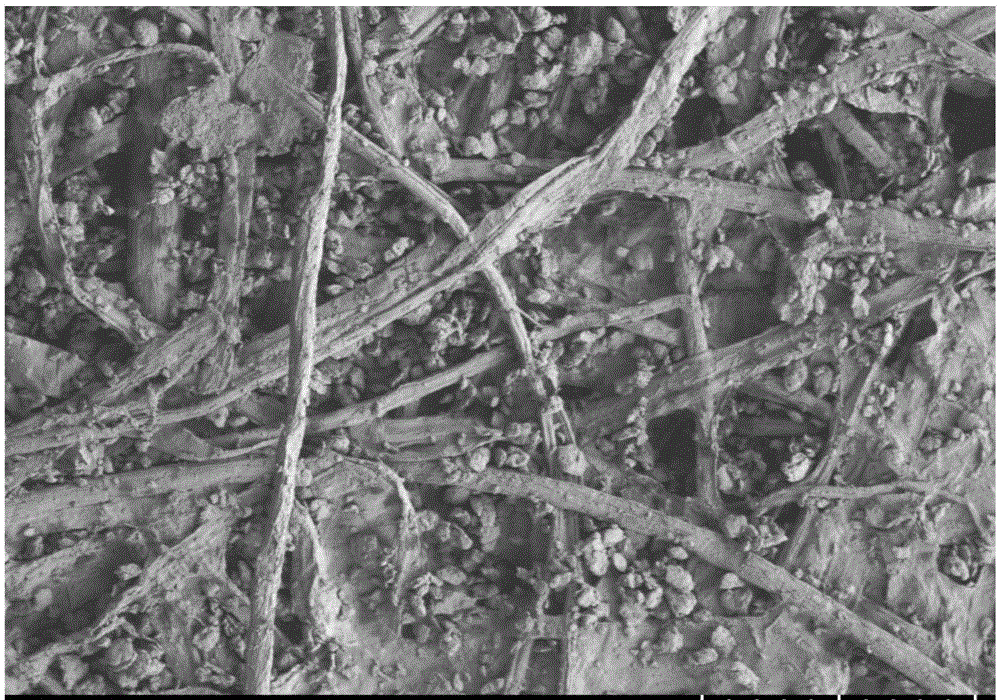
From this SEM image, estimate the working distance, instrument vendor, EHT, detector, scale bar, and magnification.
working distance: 9.8 mm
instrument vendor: Hitachi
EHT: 1 kV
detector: SE(L)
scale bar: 100000 nm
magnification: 0.35 K X